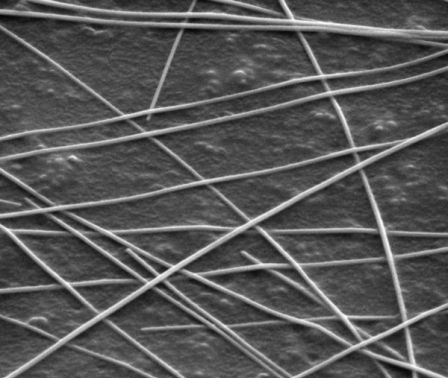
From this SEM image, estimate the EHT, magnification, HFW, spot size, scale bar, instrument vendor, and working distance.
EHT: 5 kV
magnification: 50 K X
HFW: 2.98 µm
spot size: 4.5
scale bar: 1000 nm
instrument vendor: FEI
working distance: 7.5 mm